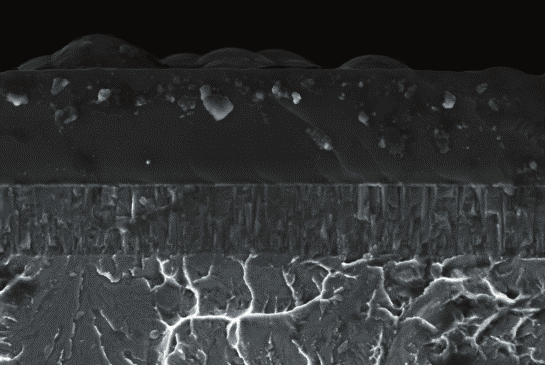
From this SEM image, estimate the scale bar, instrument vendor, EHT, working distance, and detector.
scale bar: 1000 nm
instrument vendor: Zeiss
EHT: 4 kV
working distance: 4 mm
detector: InLens